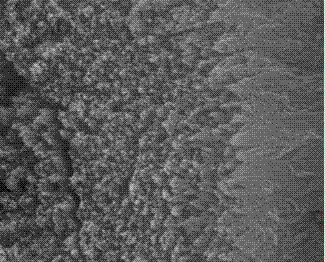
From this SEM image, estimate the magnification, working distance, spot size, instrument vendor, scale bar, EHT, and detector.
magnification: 60 K X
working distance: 11.8 mm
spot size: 4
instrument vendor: FEI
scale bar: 1000 nm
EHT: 20 kV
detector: ETD